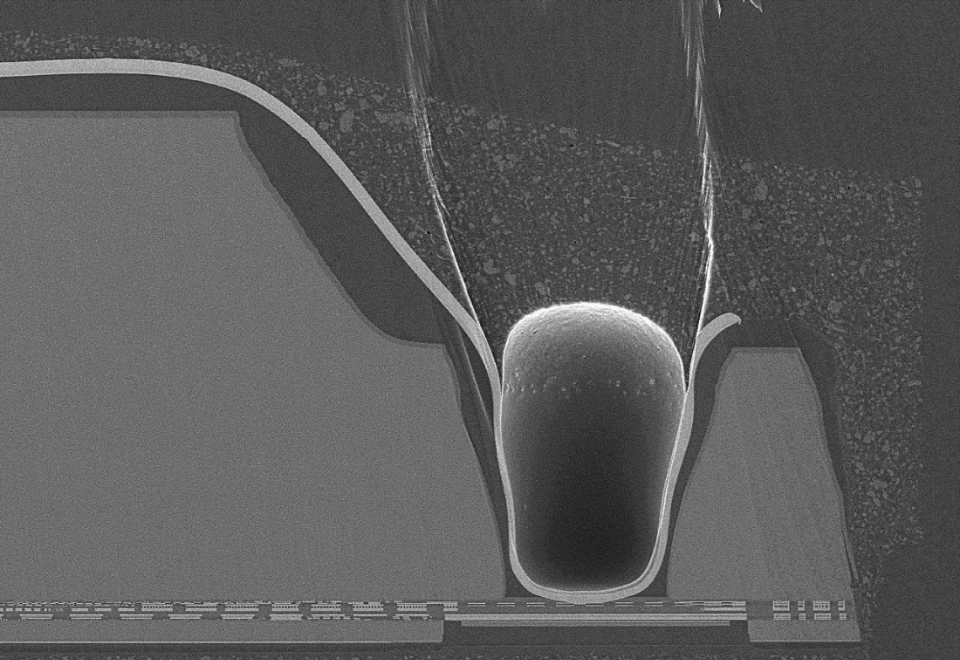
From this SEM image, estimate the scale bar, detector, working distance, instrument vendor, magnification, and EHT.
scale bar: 100000 nm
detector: LM(L)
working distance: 8 mm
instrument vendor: Hitachi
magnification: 0.45 K X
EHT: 10 kV